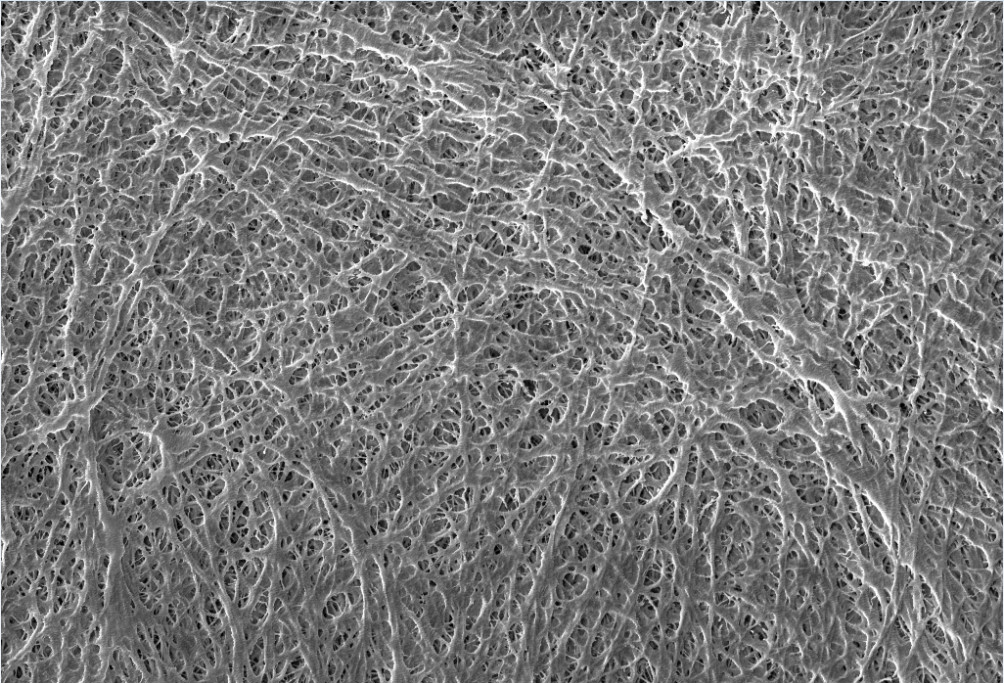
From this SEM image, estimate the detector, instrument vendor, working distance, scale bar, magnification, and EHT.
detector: SE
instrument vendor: other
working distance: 12 mm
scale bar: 3000 nm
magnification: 10 K X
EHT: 10 kV